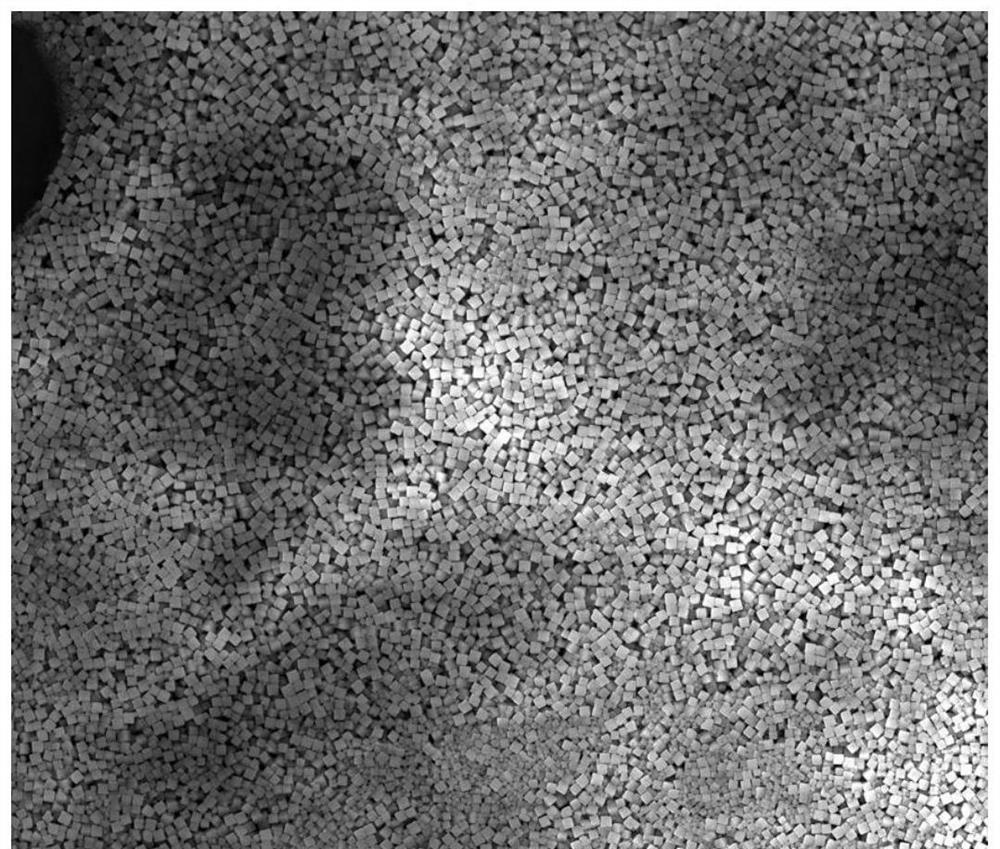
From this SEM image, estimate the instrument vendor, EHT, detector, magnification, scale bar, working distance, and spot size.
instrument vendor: FEI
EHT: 20 kV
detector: ETD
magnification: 1 K X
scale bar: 50000 nm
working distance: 10.8 mm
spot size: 4.5